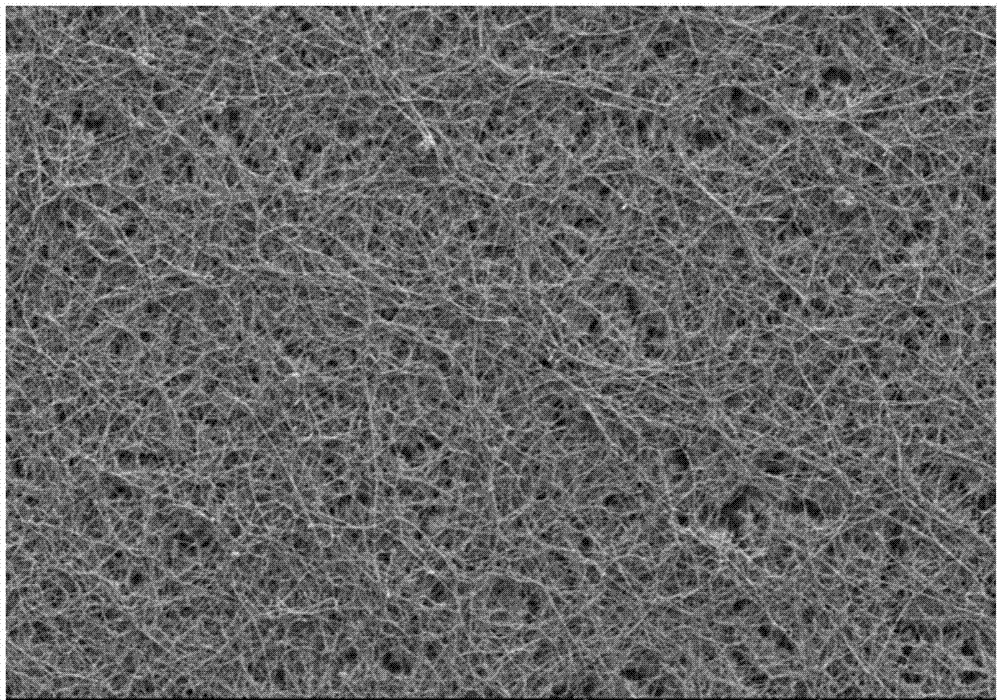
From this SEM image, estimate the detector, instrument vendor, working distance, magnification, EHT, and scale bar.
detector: SE(M)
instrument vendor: Hitachi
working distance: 8 mm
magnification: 0.5 K X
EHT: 5 kV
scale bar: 100000 nm